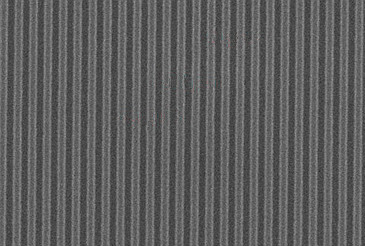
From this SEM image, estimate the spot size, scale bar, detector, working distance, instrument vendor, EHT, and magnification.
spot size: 1.5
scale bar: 1000 nm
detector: ETD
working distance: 10.5 mm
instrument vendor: FEI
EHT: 15 kV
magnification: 75 K X